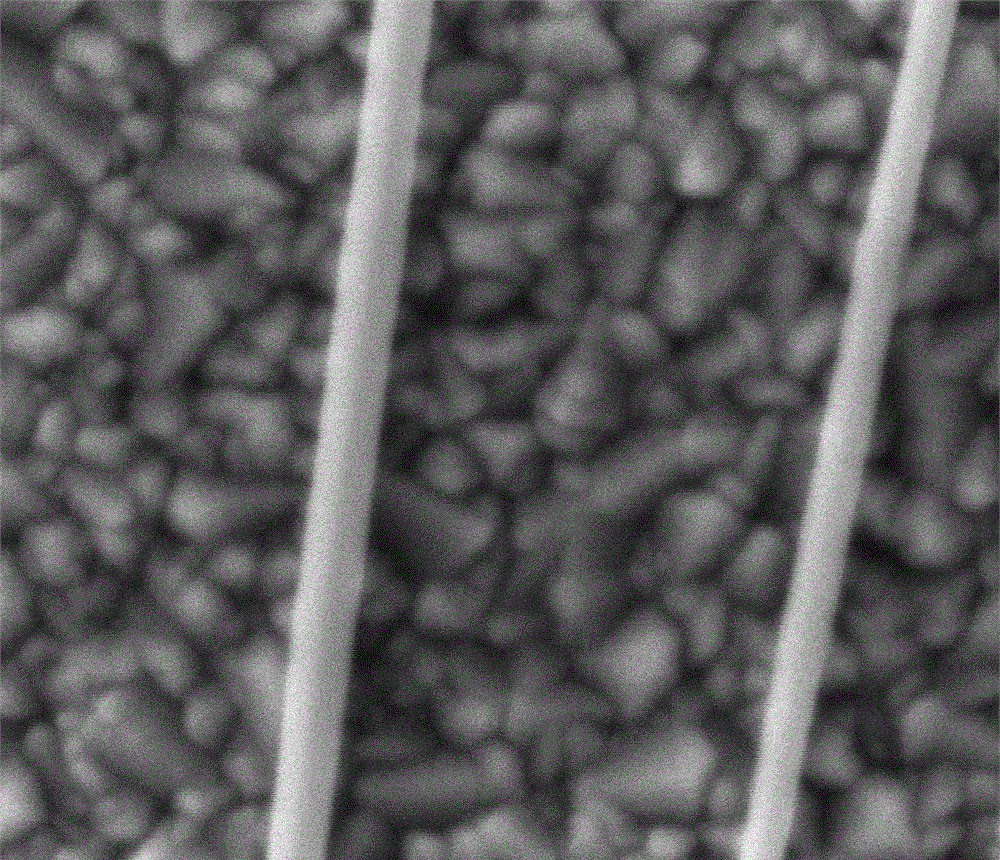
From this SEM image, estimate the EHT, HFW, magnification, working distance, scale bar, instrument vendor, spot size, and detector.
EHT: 5 kV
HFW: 1.49 µm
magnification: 100 K X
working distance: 6.8 mm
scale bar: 400 nm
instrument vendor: FEI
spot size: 2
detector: ETD